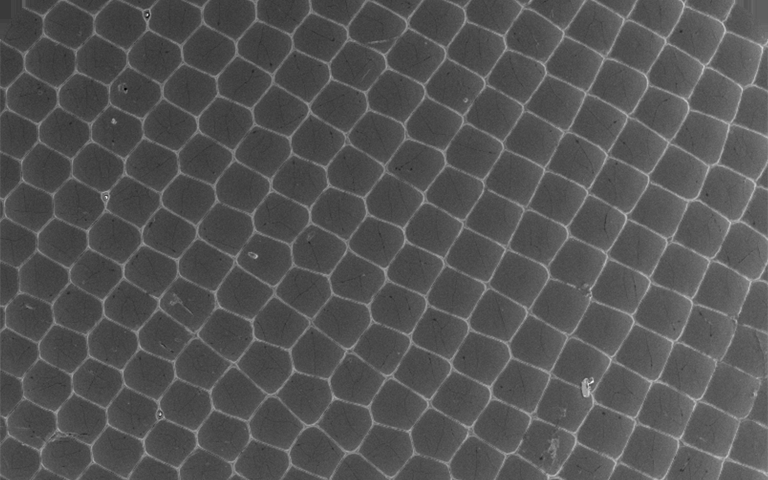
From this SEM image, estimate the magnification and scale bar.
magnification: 0.2 K X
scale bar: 100000 nm